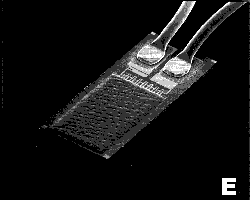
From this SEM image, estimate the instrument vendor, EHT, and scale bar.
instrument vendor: JEOL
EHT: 20 kV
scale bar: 1000000 nm